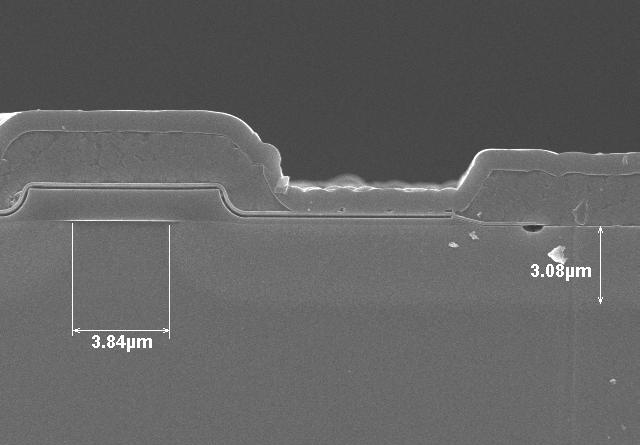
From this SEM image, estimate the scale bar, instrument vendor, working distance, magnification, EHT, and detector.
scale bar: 10000 nm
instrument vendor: Hitachi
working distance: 9.9 mm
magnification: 5 K X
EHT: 10 kV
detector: SE(U)